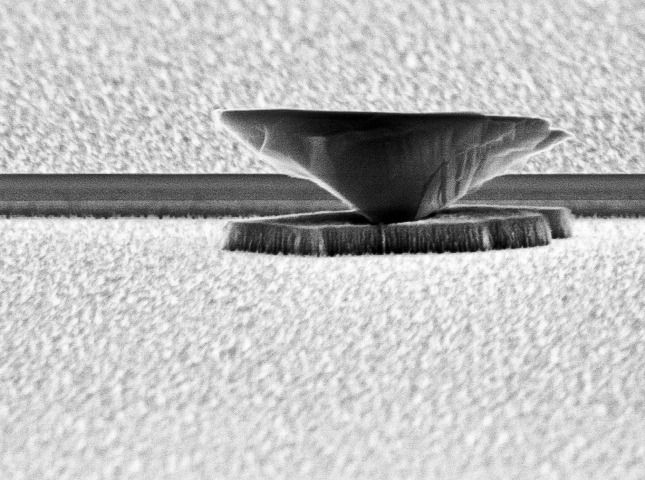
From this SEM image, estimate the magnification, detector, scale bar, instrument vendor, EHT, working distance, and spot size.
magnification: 19.151 K X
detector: SE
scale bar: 1000 nm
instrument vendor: FEI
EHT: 10 kV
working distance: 18.3 mm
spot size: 3